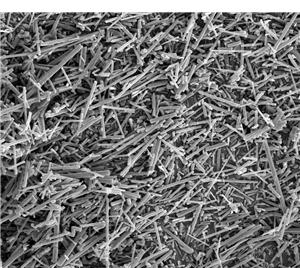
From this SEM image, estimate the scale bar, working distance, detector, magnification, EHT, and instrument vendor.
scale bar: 20000 nm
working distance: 10.7 mm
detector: ETD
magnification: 2.5 K X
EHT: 10 kV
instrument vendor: FEI